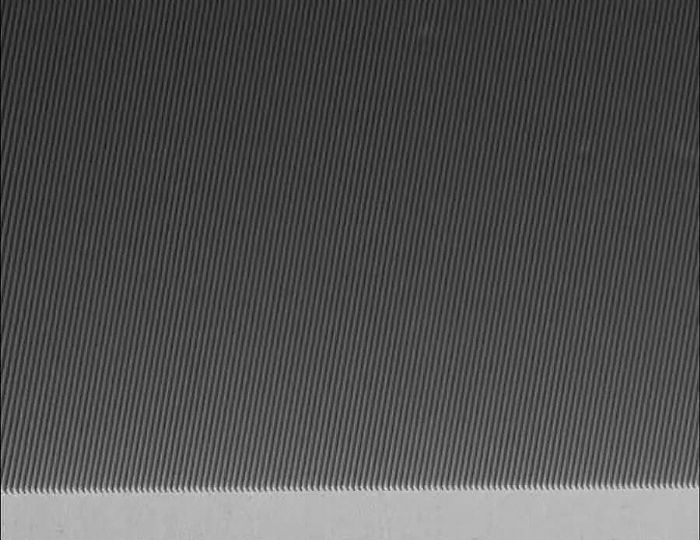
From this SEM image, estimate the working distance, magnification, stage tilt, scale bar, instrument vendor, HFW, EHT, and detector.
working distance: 4.9 mm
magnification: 5 K X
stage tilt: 30°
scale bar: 2000 nm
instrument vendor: Zeiss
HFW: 55.2 µm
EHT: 5 kV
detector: InLens